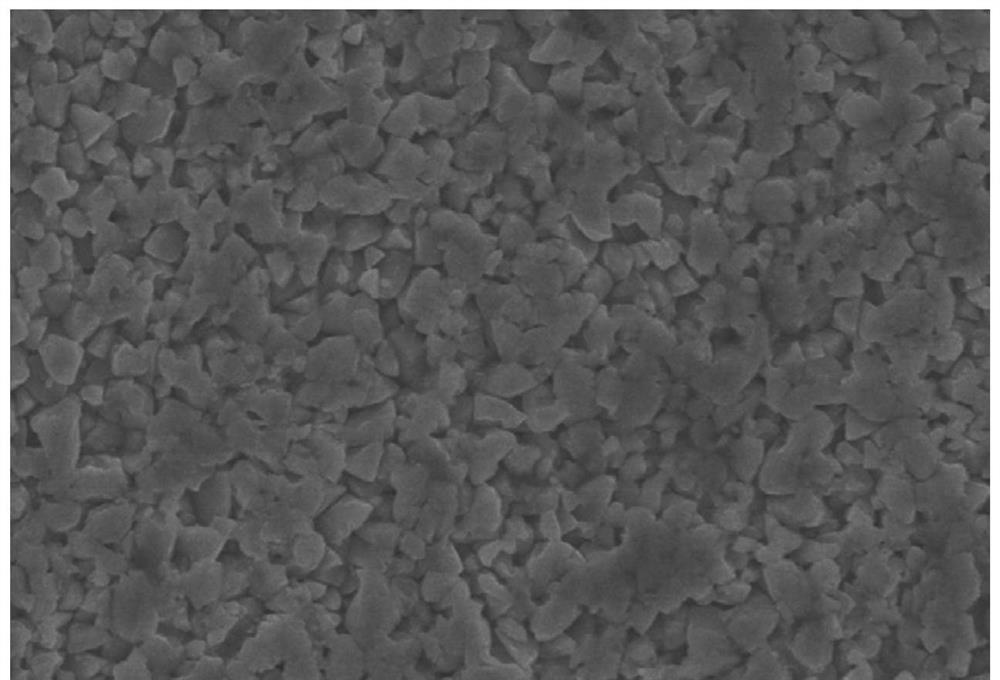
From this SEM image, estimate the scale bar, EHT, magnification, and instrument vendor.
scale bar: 10000 nm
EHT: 20 kV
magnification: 2 K X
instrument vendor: other